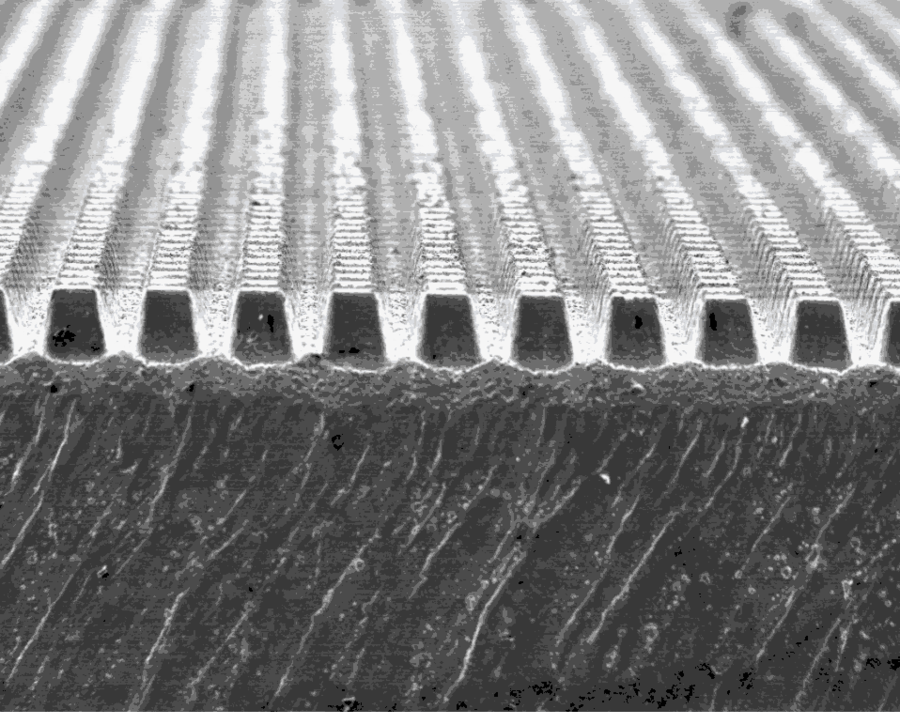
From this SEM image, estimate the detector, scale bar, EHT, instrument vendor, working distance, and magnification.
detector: ETD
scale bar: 1000000 nm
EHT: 20 kV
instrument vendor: FEI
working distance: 11.6 mm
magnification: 0.05 K X